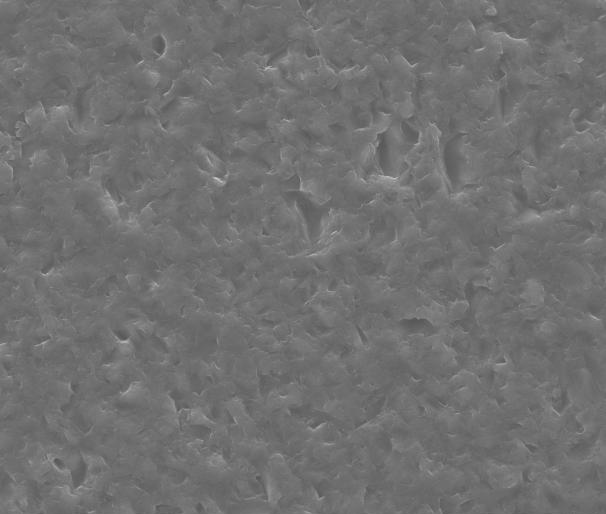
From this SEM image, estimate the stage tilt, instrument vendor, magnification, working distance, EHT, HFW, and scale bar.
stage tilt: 0°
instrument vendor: FEI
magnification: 2 K X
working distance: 11.9 mm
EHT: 25 kV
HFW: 135 µm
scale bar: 50000 nm